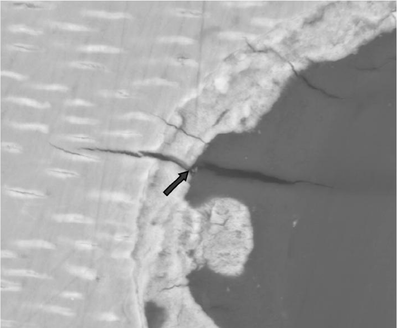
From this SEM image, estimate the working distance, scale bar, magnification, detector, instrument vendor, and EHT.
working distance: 10.3 mm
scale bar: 50000 nm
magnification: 2 K X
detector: SSD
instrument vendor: FEI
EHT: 30 kV